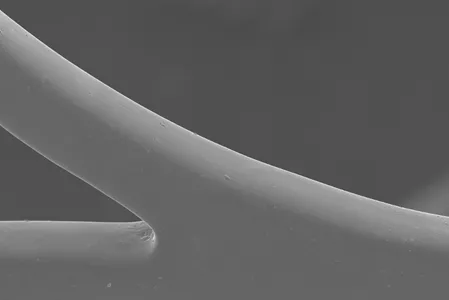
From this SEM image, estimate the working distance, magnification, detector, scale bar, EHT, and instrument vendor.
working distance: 11 mm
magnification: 0.3 K X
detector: SE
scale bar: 100000 nm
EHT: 15 kV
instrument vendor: Hitachi